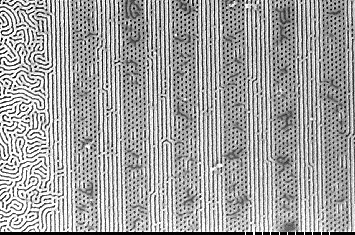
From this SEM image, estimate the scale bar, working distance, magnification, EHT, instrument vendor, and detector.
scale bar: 1000 nm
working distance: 3.2 mm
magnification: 30 K X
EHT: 1 kV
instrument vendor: Hitachi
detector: SE(U, _A0)